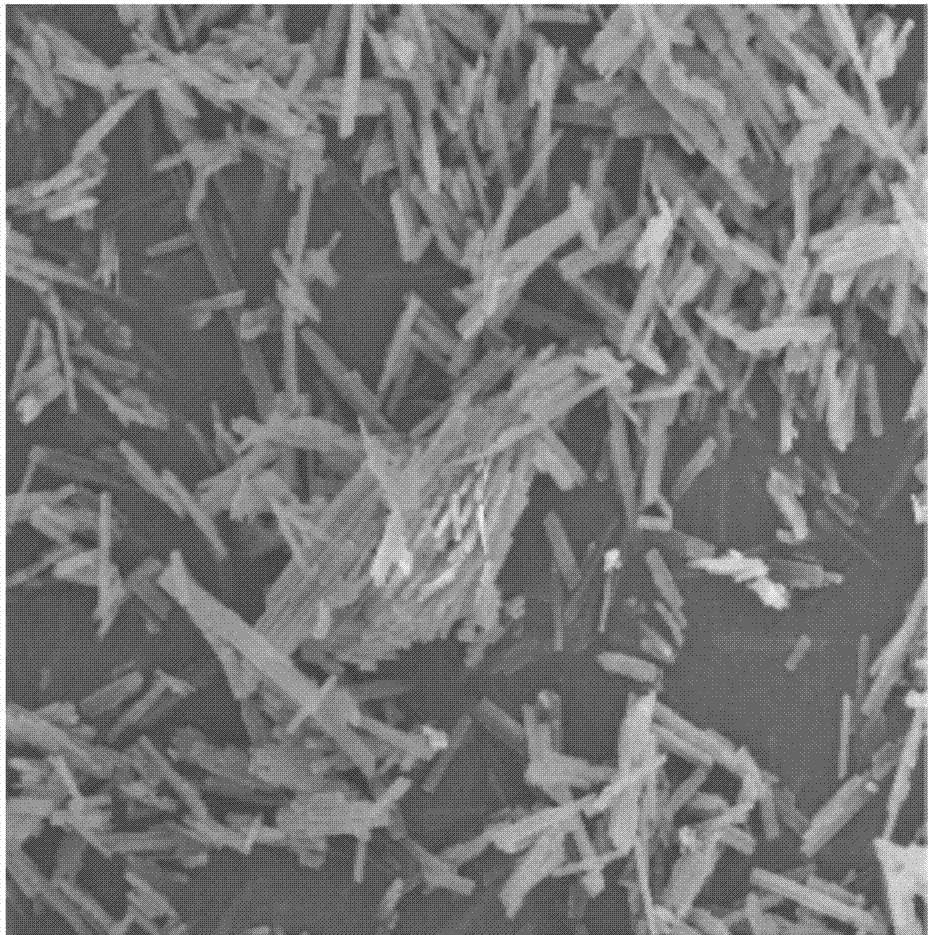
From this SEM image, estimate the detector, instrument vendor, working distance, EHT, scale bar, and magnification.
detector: SEI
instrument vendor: JEOL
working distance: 6 mm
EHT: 10 kV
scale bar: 10000 nm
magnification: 2 K X